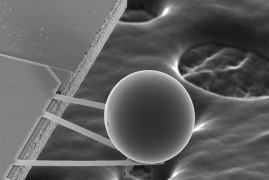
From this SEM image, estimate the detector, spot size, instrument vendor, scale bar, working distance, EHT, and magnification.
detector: SE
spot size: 6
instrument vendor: FEI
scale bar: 100000 nm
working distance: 34.4 mm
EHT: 20 kV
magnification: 0.384 K X